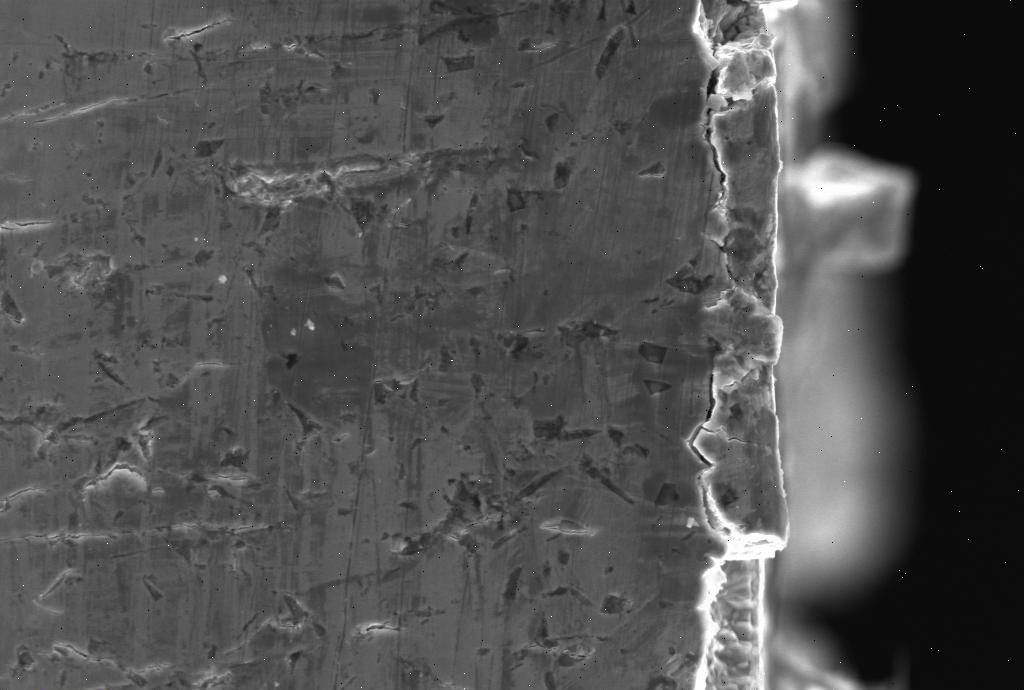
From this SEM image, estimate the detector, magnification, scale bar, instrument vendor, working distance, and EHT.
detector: SE1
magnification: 2 K X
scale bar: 10000 nm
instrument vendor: Zeiss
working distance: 30 mm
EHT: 20 kV